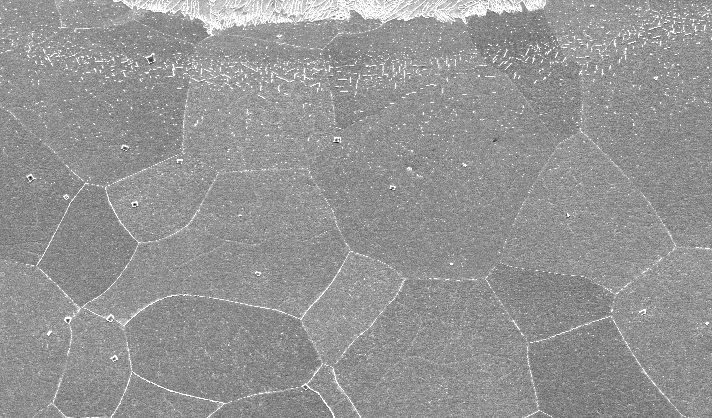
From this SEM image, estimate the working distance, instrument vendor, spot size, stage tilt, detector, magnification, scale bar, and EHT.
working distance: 10.5 mm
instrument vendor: FEI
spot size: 5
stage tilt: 0°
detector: SE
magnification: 0.2 K X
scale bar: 100000 nm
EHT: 20 kV